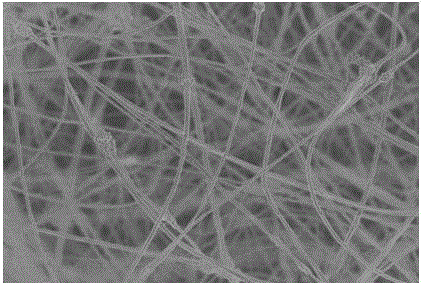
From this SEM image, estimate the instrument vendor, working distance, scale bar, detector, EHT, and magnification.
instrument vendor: Hitachi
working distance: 8.4 mm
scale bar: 10000 nm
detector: SE(UL)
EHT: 3 kV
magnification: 3 K X